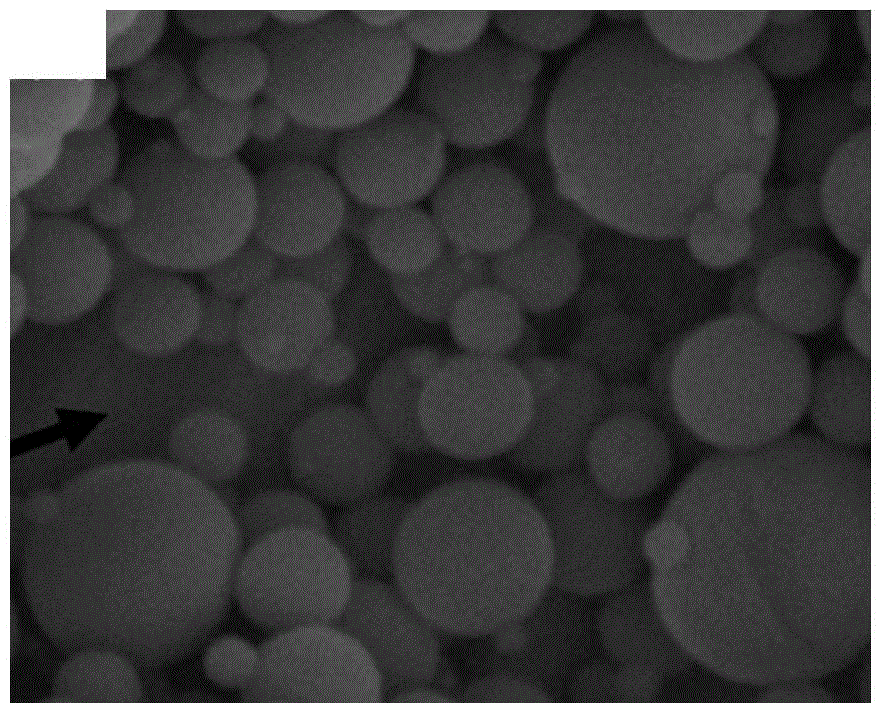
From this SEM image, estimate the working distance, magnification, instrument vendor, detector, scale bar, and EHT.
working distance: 5.4 mm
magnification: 100 K X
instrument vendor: FEI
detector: TLD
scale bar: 400 nm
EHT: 5 kV